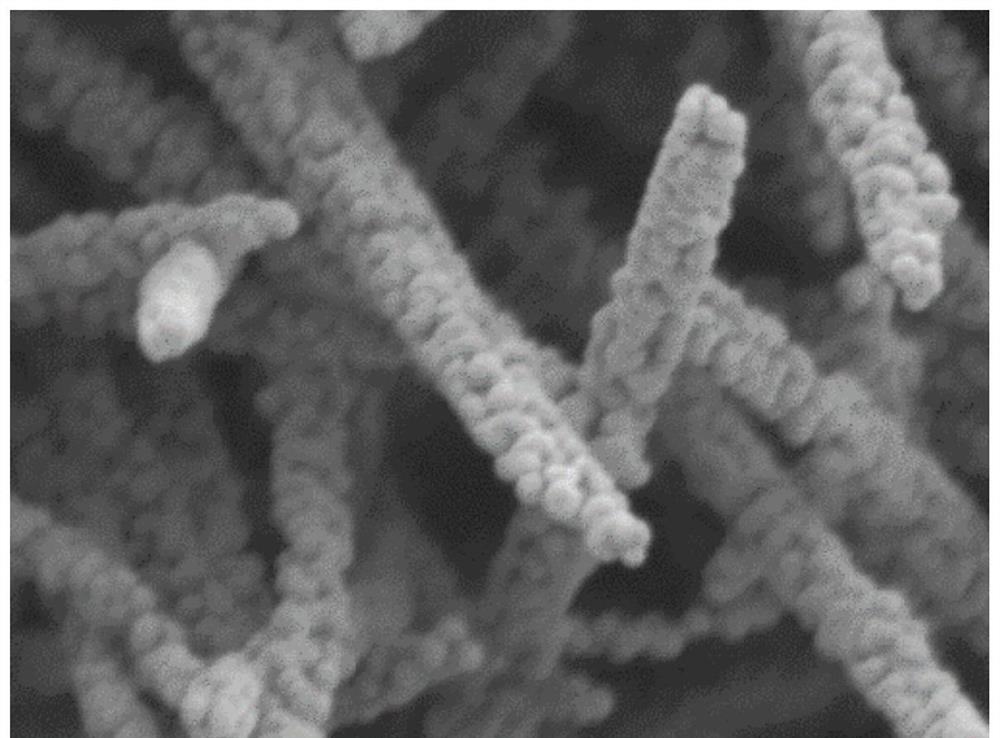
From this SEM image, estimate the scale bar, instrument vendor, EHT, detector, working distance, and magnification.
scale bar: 100 nm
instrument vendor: JEOL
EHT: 3 kV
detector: LED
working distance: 8 mm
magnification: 60 K X